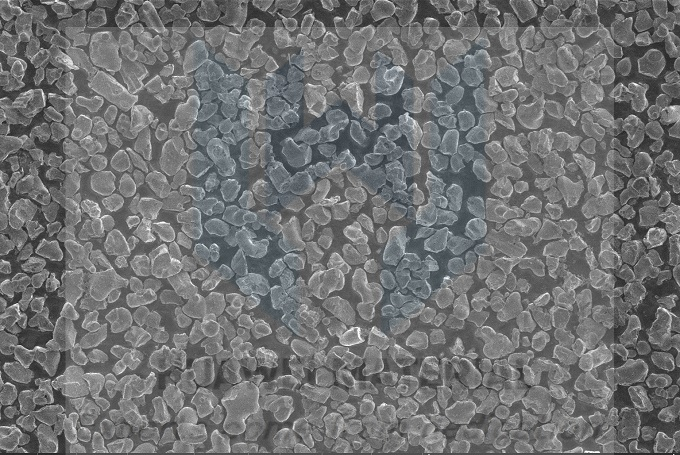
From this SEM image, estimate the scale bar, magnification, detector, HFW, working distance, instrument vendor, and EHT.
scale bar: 30000 nm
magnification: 5 K X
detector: ETD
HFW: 82.9 µm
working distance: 4.3 mm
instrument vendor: FEI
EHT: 2 kV